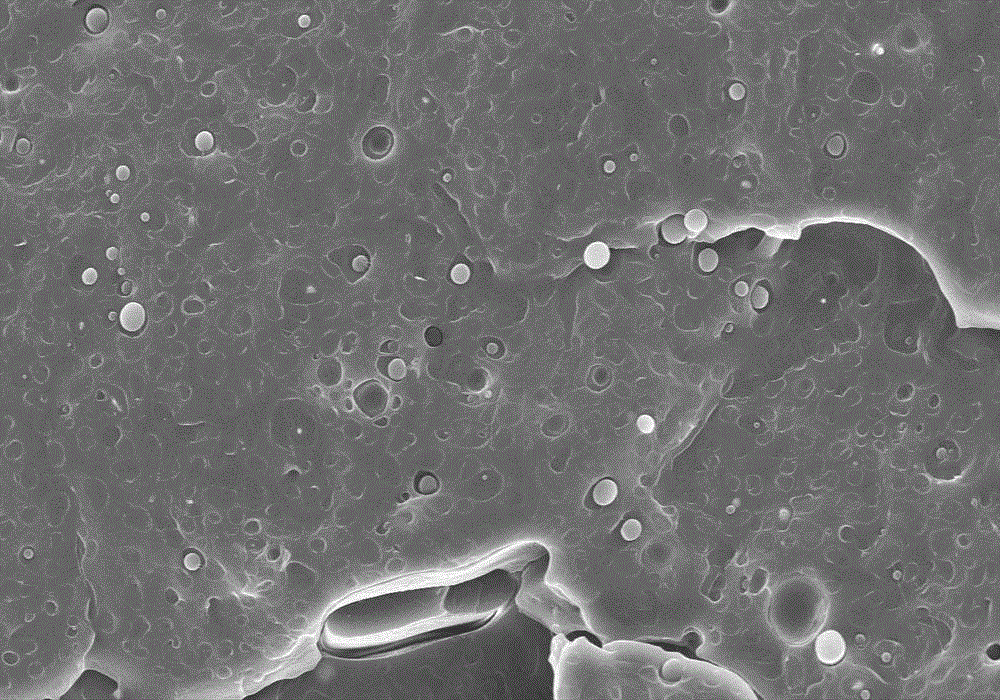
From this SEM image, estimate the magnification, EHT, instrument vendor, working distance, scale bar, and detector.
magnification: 2 K X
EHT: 10 kV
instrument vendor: Hitachi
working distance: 10.8 mm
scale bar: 20000 nm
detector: SE(M)